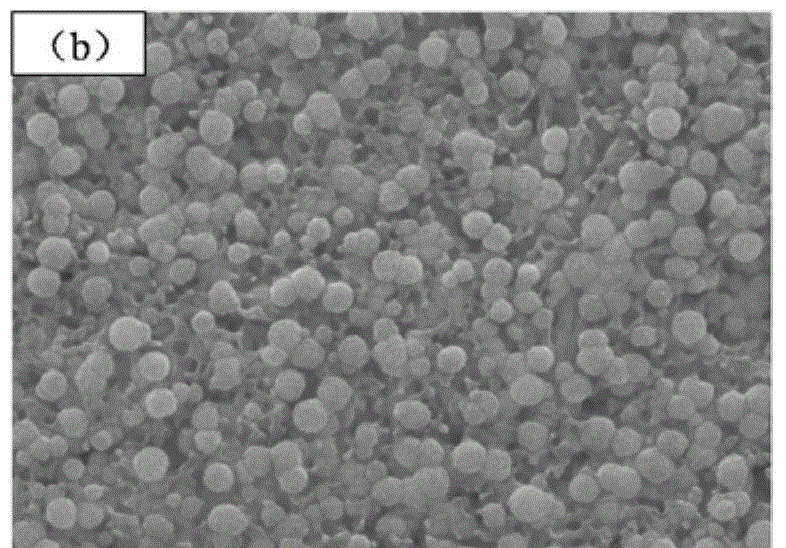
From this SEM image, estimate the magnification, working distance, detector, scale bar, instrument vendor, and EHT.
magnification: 50 K X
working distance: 7.2 mm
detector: SEI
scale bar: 100 nm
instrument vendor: JEOL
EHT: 15 kV